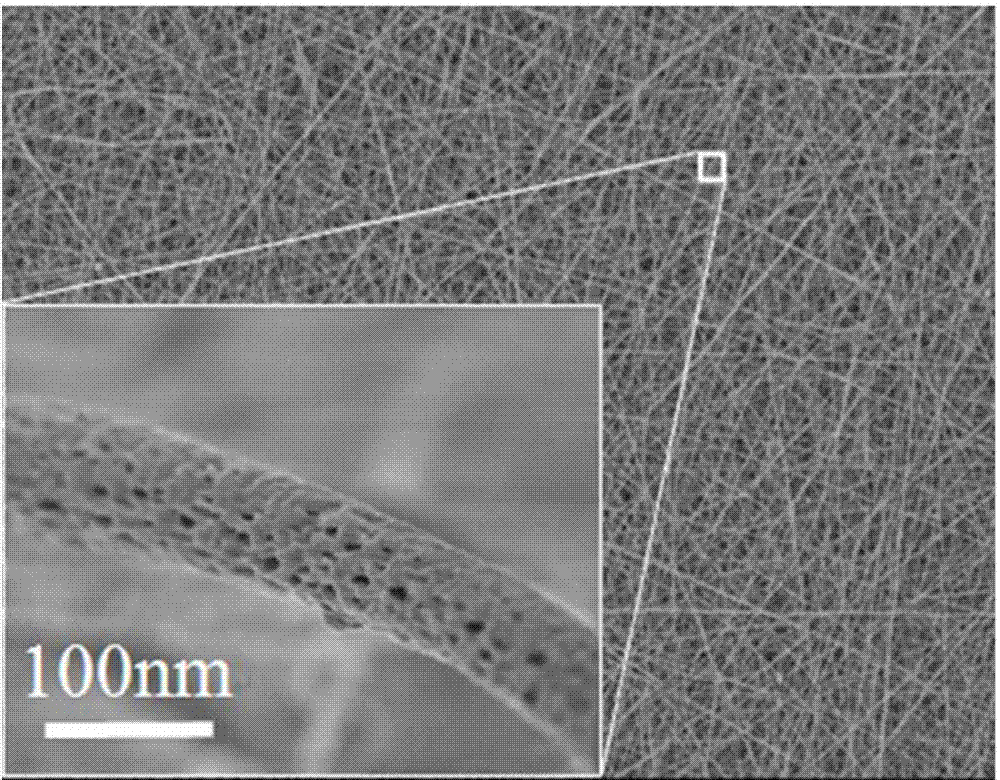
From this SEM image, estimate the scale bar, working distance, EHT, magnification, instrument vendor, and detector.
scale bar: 10000 nm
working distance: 13 mm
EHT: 5 kV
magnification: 2 K X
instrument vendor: JEOL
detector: LEI